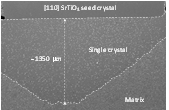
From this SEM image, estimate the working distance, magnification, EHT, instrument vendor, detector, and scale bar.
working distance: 12.2 mm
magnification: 0.05 K X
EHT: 15 kV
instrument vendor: Hitachi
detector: SE(U)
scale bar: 1000000 nm